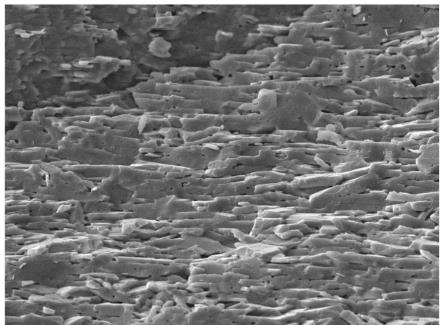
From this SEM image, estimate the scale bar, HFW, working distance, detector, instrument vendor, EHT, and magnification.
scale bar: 20000 nm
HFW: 138 µm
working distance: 11.14 mm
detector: SE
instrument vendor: Tescan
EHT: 20 kV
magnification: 2 K X